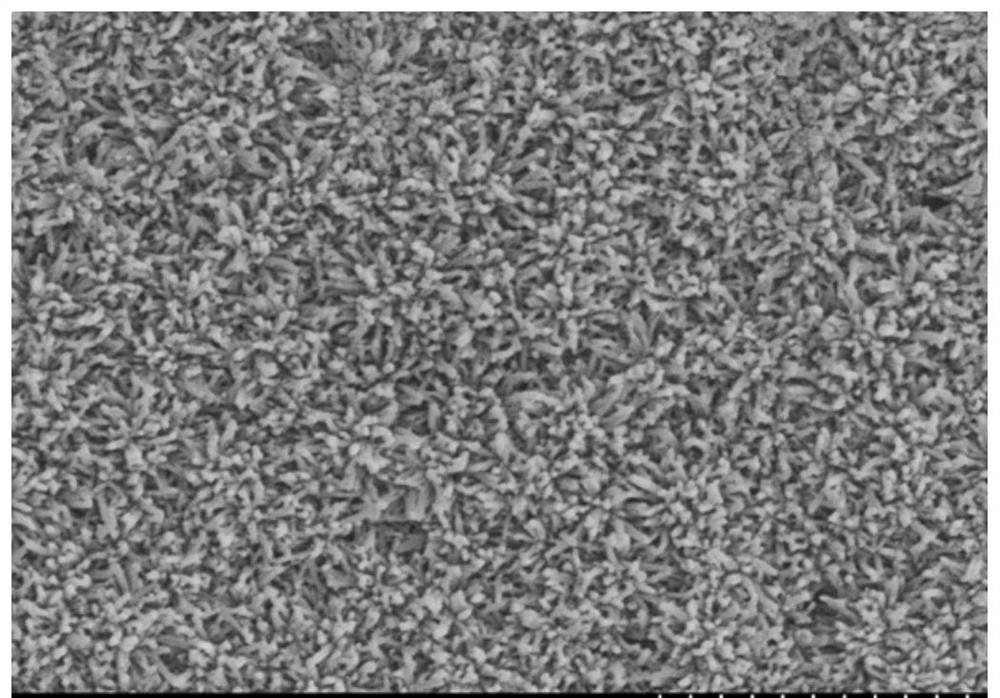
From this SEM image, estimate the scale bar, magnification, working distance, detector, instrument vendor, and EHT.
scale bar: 2000 nm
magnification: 20 K X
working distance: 9.5 mm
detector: SE(M)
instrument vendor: Hitachi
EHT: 3 kV